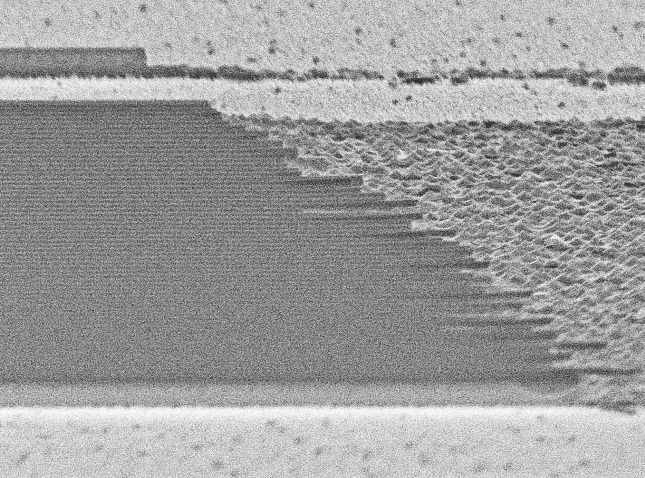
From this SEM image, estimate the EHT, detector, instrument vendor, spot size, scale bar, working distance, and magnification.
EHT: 10 kV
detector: SE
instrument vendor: FEI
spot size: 3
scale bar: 2000 nm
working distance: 23.2 mm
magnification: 11.81 K X